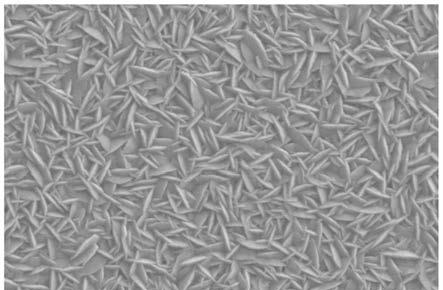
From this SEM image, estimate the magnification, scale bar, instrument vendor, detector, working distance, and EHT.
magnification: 40 K X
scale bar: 200 nm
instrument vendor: Zeiss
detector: SE2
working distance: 3.4 mm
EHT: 3 kV